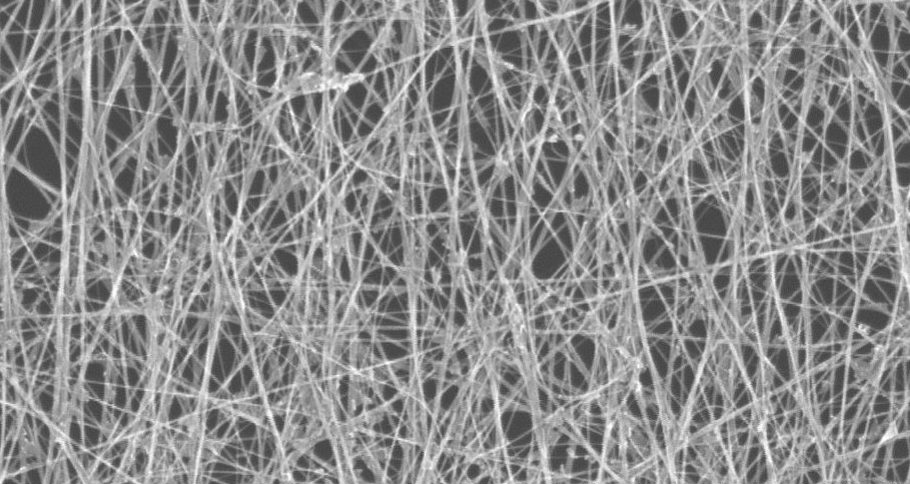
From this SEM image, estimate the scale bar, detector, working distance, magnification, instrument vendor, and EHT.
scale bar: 1000 nm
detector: SE(U)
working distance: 6.4 mm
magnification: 50 K X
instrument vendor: Hitachi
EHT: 15 kV